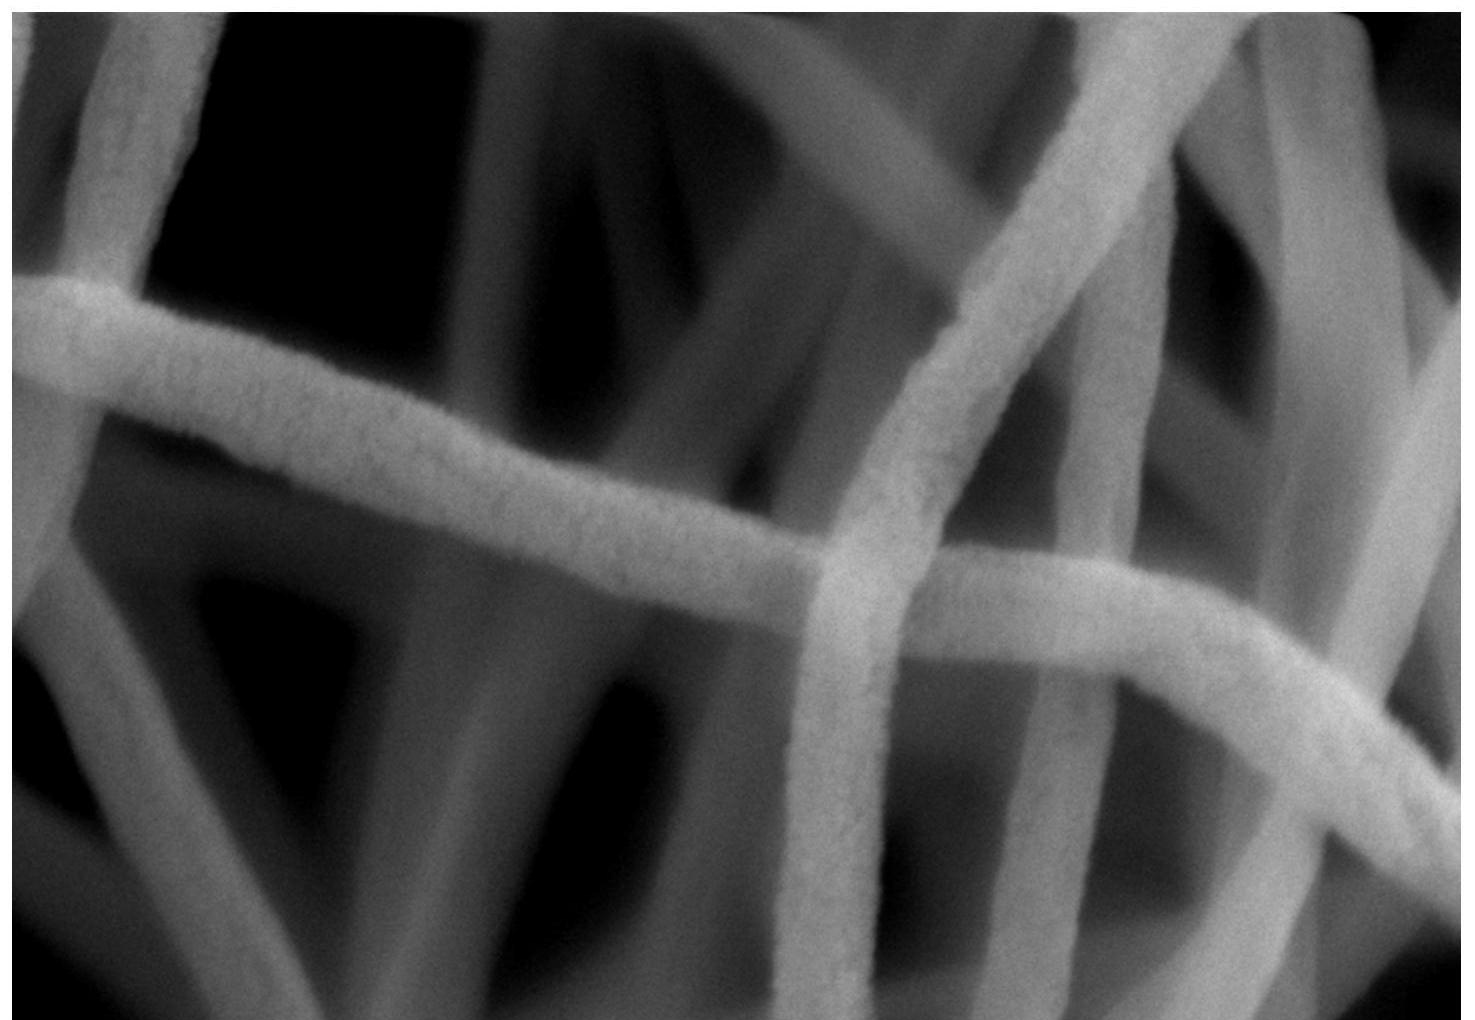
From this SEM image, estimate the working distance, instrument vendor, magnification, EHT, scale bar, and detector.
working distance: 8.6 mm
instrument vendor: Zeiss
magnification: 100 K X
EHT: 10 kV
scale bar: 100 nm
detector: SE2